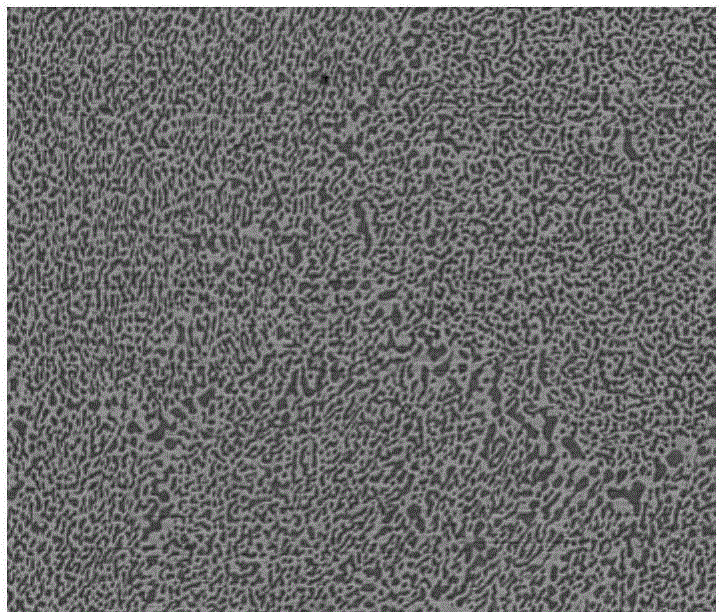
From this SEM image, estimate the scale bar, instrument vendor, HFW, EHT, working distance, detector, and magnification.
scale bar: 50000 nm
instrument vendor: FEI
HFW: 149 µm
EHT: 20 kV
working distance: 9.7 mm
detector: BSED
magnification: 2 K X